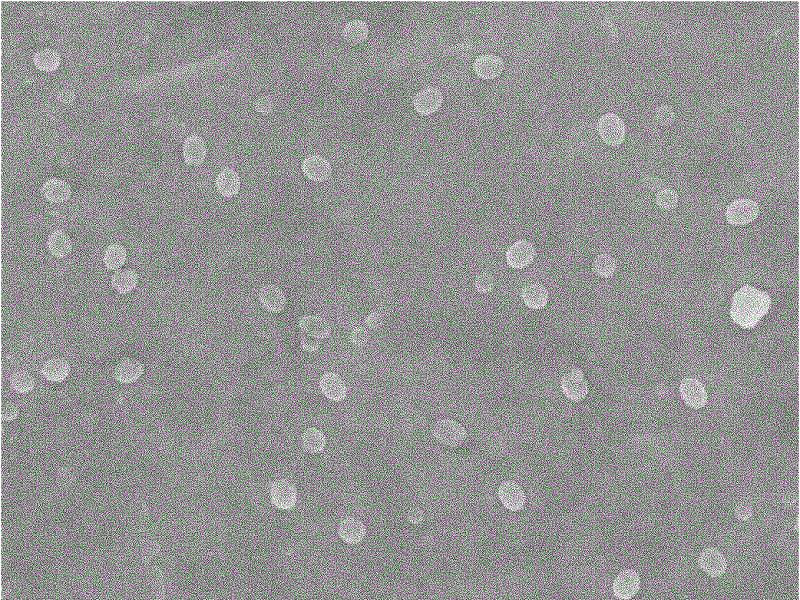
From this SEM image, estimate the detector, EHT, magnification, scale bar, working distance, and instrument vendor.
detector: SEI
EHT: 5 kV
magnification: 10 K X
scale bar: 1000 nm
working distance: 6.1 mm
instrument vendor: JEOL